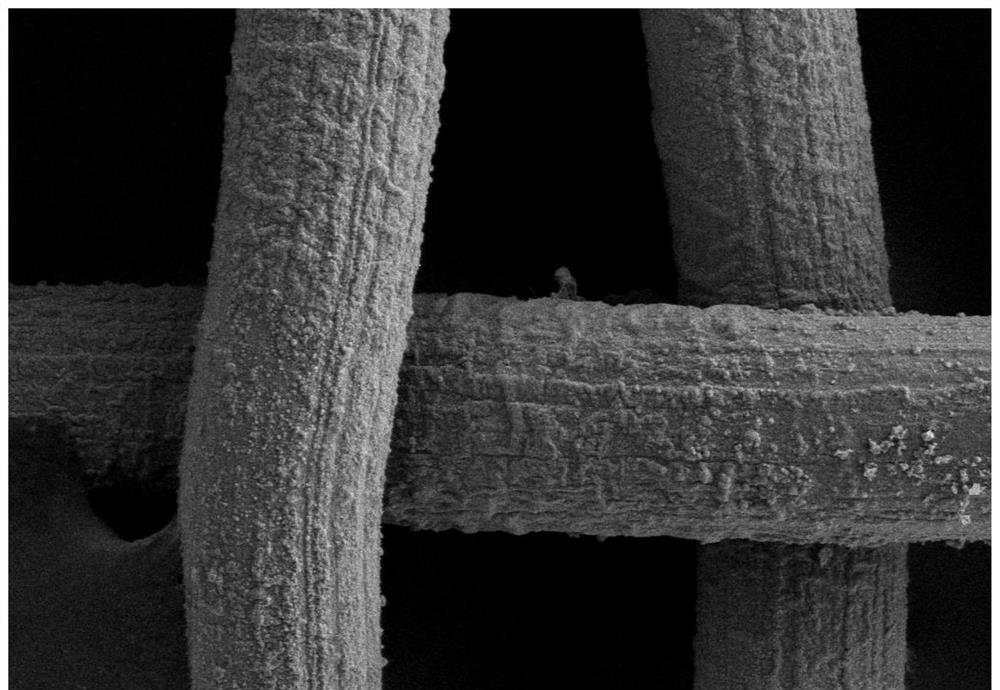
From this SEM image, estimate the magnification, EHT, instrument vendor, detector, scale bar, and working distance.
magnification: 0.5 K X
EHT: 10 kV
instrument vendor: Zeiss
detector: SE2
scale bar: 10000 nm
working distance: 7.2 mm